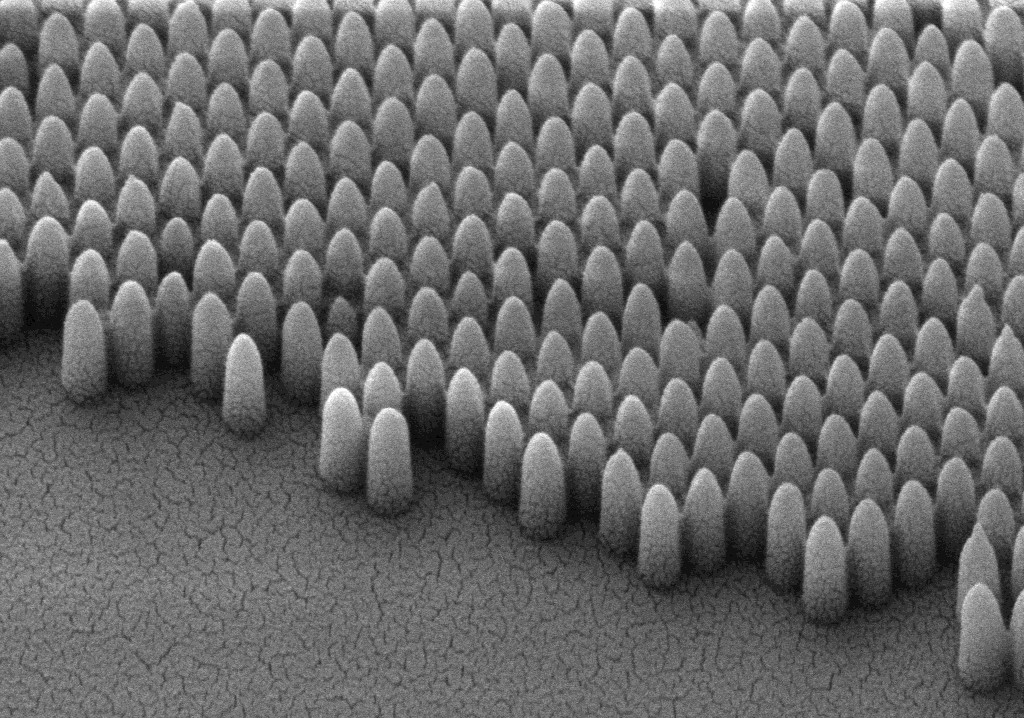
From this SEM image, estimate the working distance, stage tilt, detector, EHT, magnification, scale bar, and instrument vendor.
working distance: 5 mm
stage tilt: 45°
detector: SE2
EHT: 5 kV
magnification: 68.82 K X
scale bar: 200 nm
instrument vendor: Zeiss